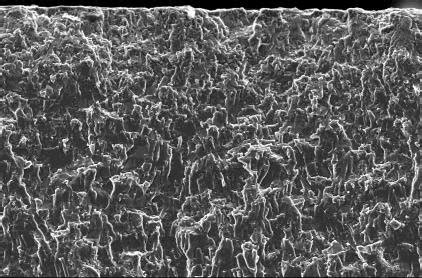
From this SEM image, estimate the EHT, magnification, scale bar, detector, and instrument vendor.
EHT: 20 kV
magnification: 0.2 K X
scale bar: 100000 nm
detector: SEI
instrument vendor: JEOL